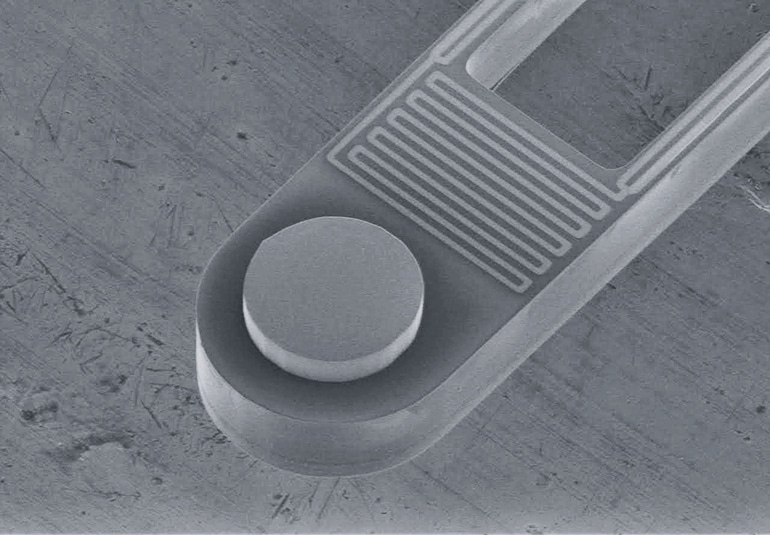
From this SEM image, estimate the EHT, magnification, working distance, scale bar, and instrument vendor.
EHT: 5 kV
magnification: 1.2 K X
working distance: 21.7 mm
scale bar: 100000 nm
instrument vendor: Hitachi